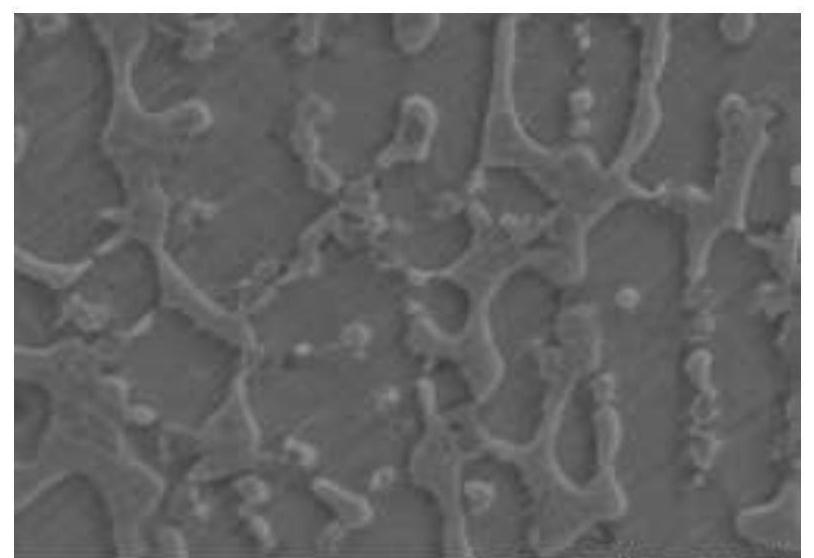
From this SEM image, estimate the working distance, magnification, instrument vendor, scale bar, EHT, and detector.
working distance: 16.2 mm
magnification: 5 K X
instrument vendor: Hitachi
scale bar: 10000 nm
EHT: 15 kV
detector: SE(UL)